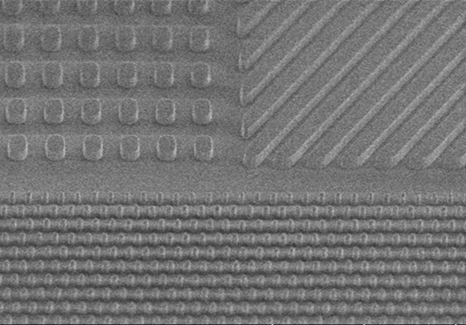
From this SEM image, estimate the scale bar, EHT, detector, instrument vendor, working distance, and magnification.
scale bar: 5000 nm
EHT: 1 kV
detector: SE(UL)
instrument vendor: Hitachi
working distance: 8.2 mm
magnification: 10 K X